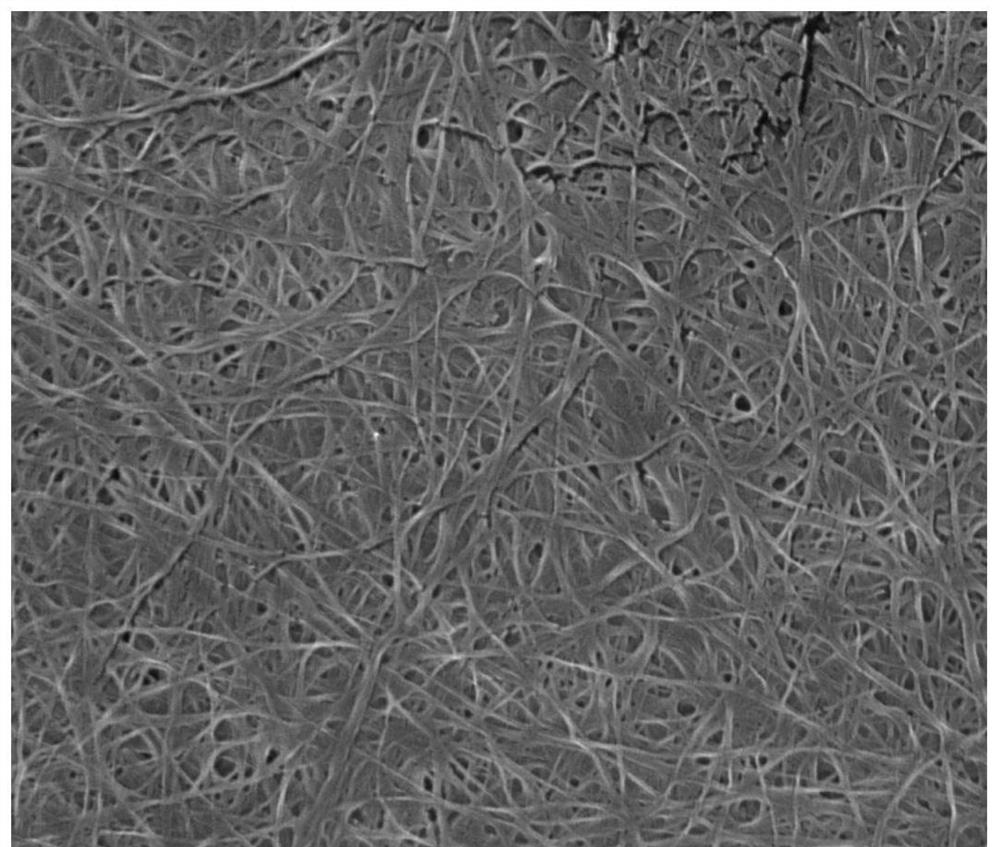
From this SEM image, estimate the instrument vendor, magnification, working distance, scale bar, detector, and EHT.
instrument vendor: FEI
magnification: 40 K X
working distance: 5.1 mm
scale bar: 3000 nm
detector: TLD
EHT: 15 kV